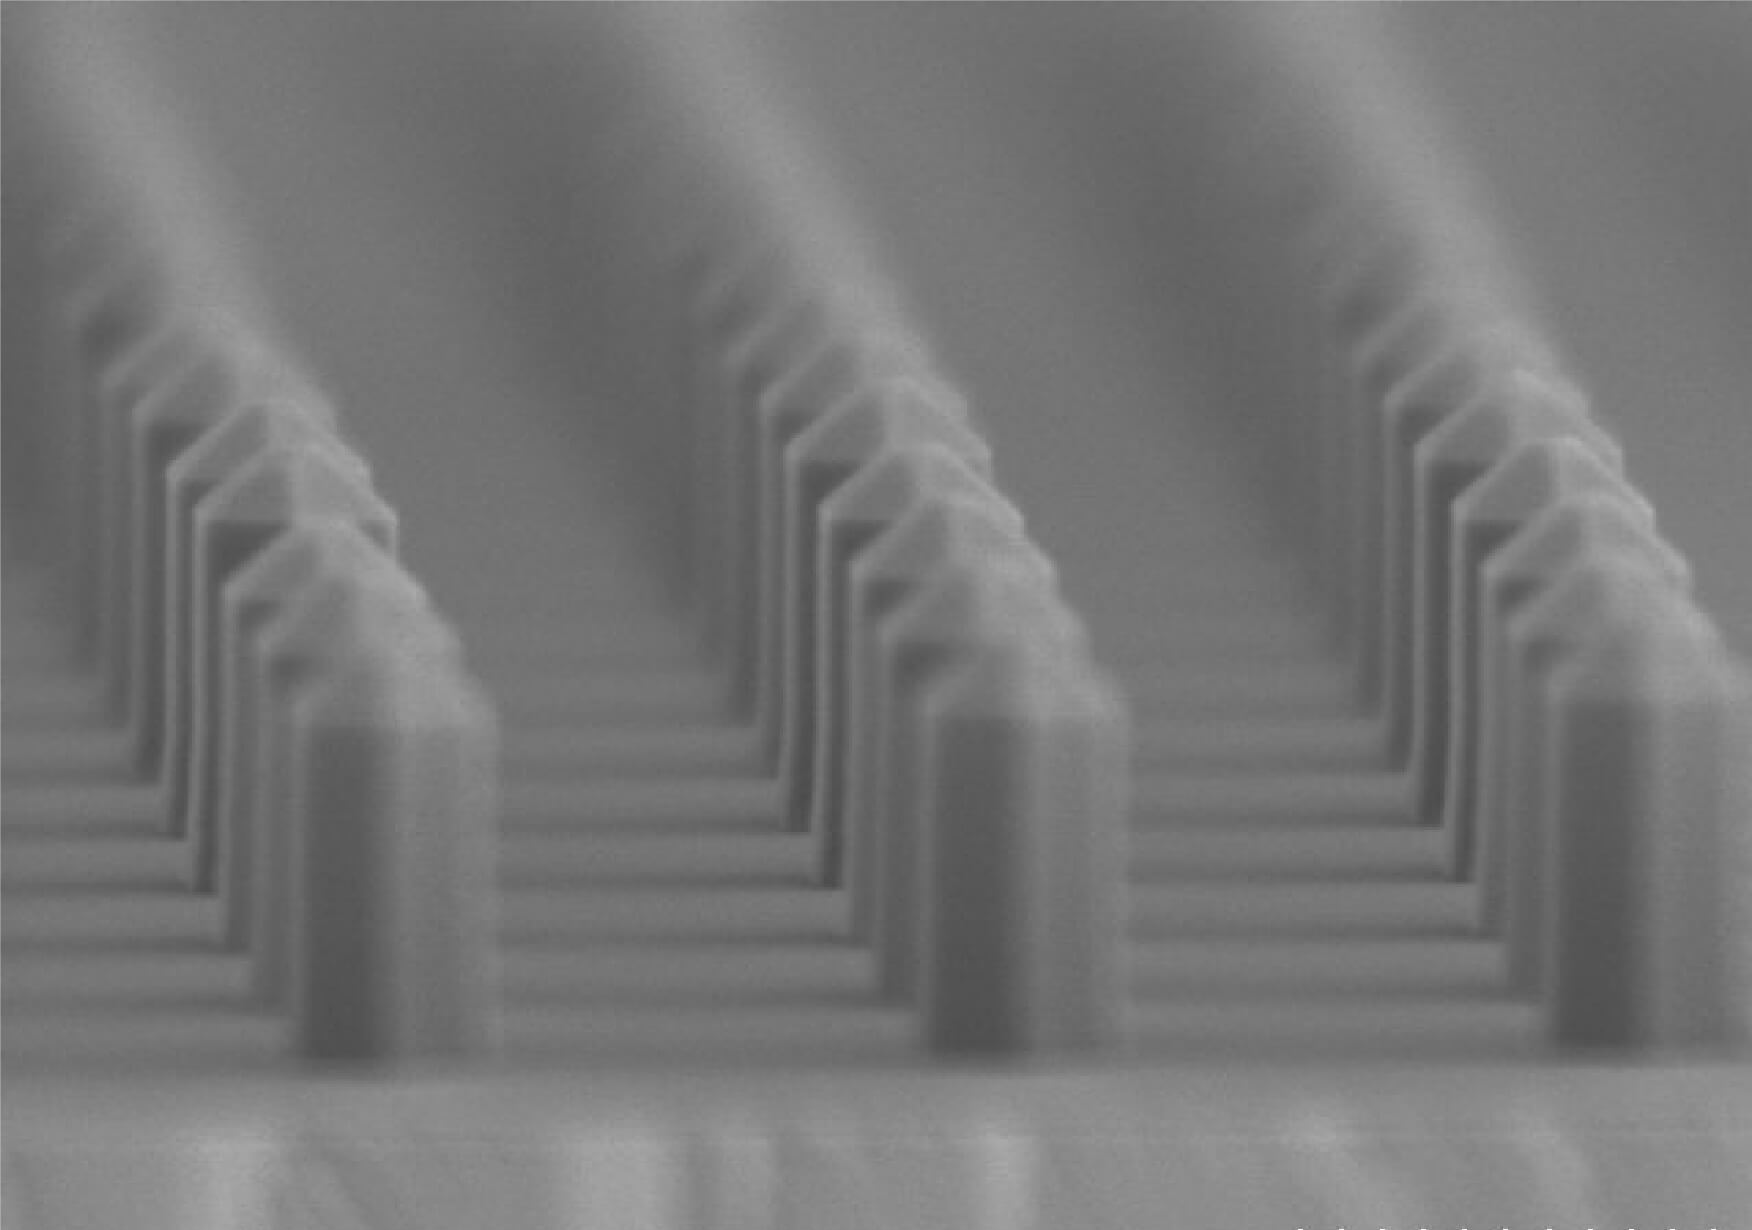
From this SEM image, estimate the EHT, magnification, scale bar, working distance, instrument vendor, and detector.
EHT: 3 kV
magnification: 30 K X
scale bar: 1000 nm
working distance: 9.4 mm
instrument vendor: Hitachi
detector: SE(M)